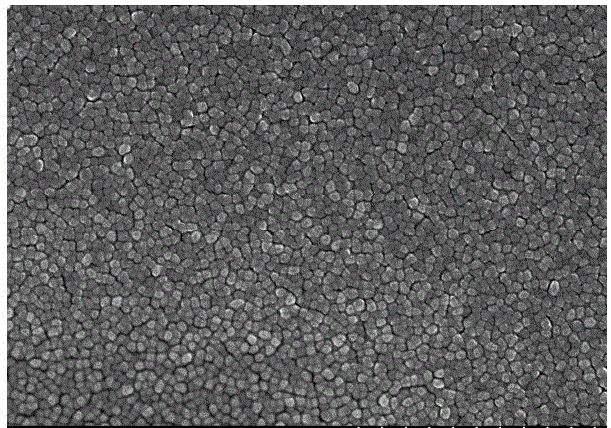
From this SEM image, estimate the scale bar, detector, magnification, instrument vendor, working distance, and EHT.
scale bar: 1000 nm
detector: SE(M)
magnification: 50 K X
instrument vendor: Hitachi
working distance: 8.1 mm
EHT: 15 kV